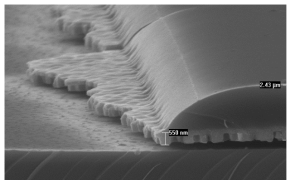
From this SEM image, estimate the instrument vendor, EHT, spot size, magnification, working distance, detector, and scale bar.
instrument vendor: FEI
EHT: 10 kV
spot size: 3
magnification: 10 K X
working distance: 20.5 mm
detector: SE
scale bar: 2000 nm